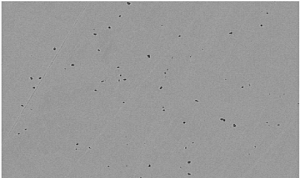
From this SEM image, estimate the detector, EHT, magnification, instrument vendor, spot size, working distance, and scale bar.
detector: BSED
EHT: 25 kV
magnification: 1 K X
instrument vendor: FEI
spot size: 4.5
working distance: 10.2 mm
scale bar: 100000 nm